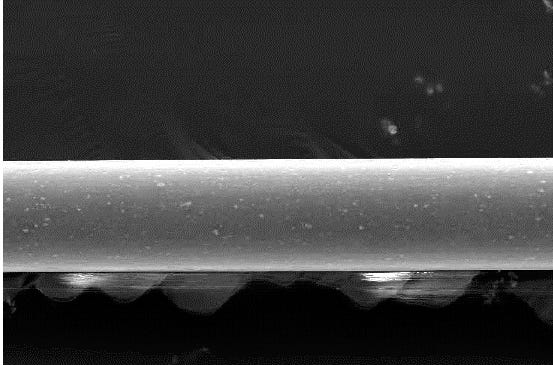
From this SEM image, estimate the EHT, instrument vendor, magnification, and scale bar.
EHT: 20 kV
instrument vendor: JEOL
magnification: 0.5 K X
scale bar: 50000 nm